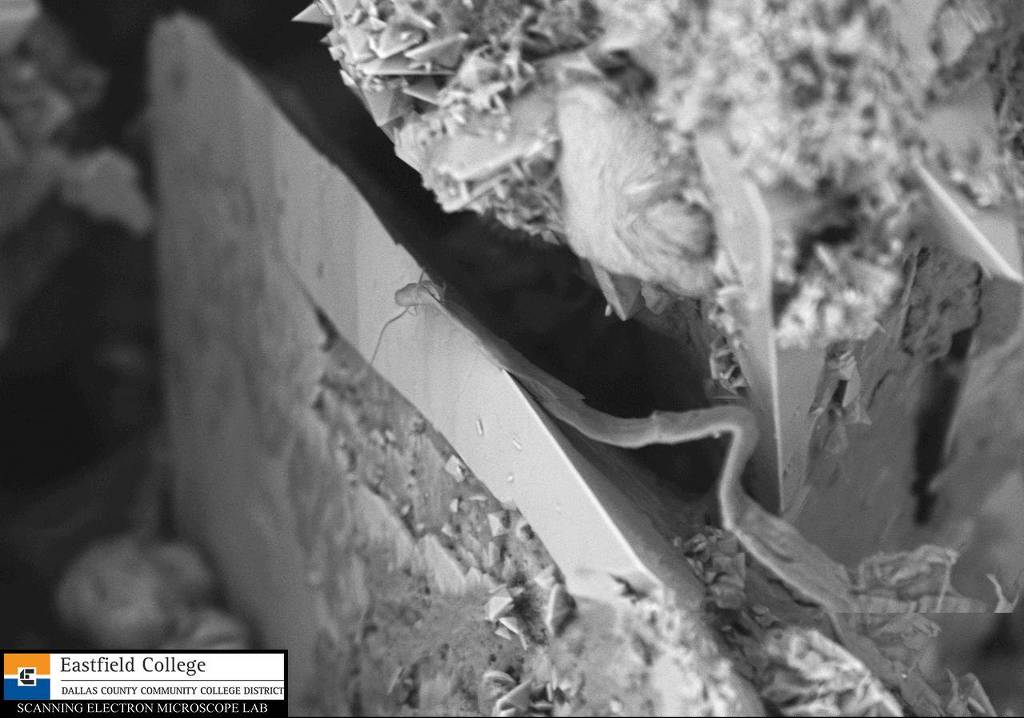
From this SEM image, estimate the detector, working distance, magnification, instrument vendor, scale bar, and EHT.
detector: BSE3D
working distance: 4.8 mm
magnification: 0.85 K X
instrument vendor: Hitachi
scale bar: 50000 nm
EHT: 10 kV